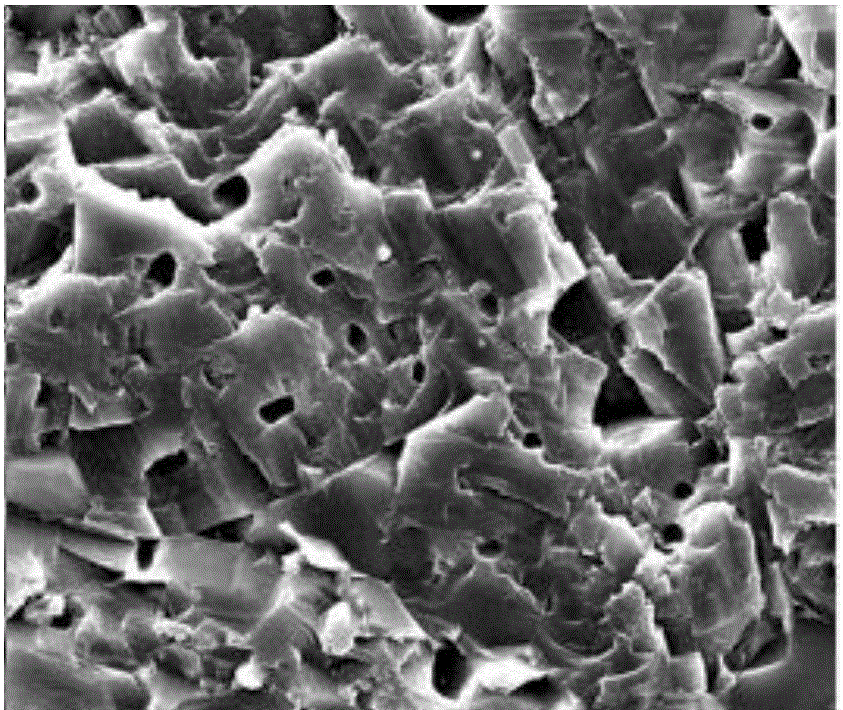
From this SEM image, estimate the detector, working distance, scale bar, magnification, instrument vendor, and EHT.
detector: SE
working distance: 9.9 mm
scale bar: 20000 nm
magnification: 5 K X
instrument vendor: FEI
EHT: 20 kV